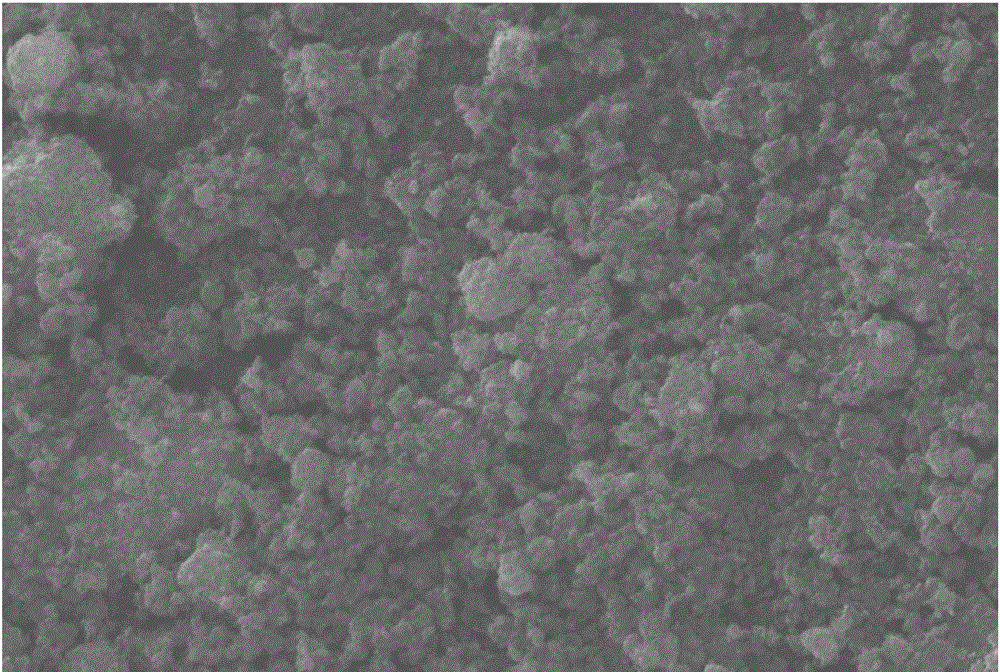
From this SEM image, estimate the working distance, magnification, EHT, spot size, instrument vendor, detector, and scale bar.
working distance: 10 mm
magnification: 5 K X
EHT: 30 kV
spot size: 25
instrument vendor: JEOL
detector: SEI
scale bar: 5000 nm